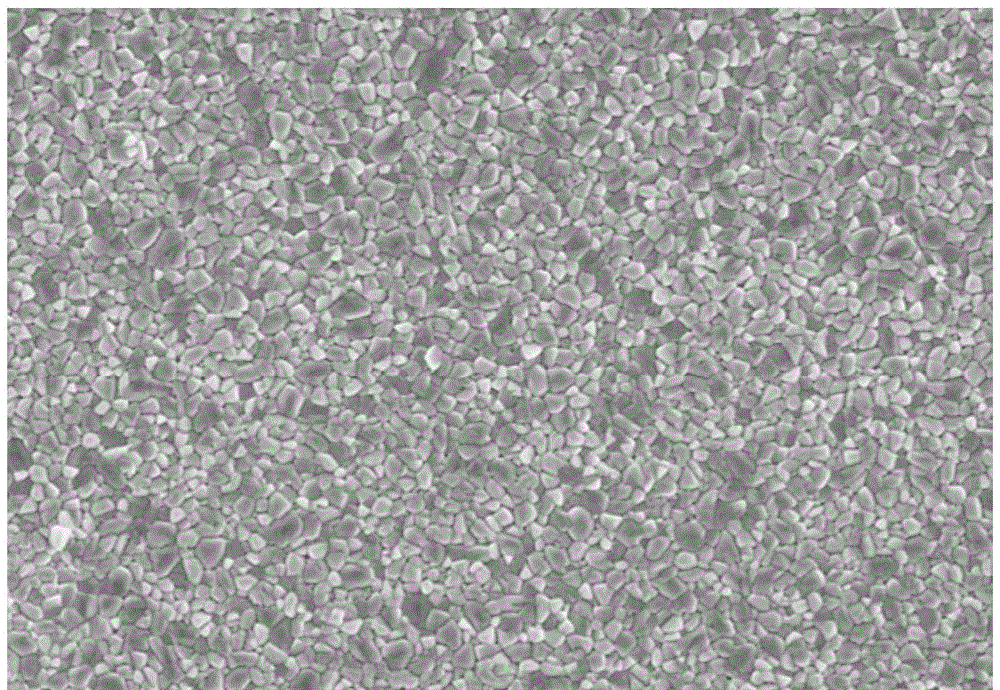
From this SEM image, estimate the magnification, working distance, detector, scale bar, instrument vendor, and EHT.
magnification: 10 K X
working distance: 8.2 mm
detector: SE(UL)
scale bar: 5000 nm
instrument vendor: Hitachi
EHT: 5 kV